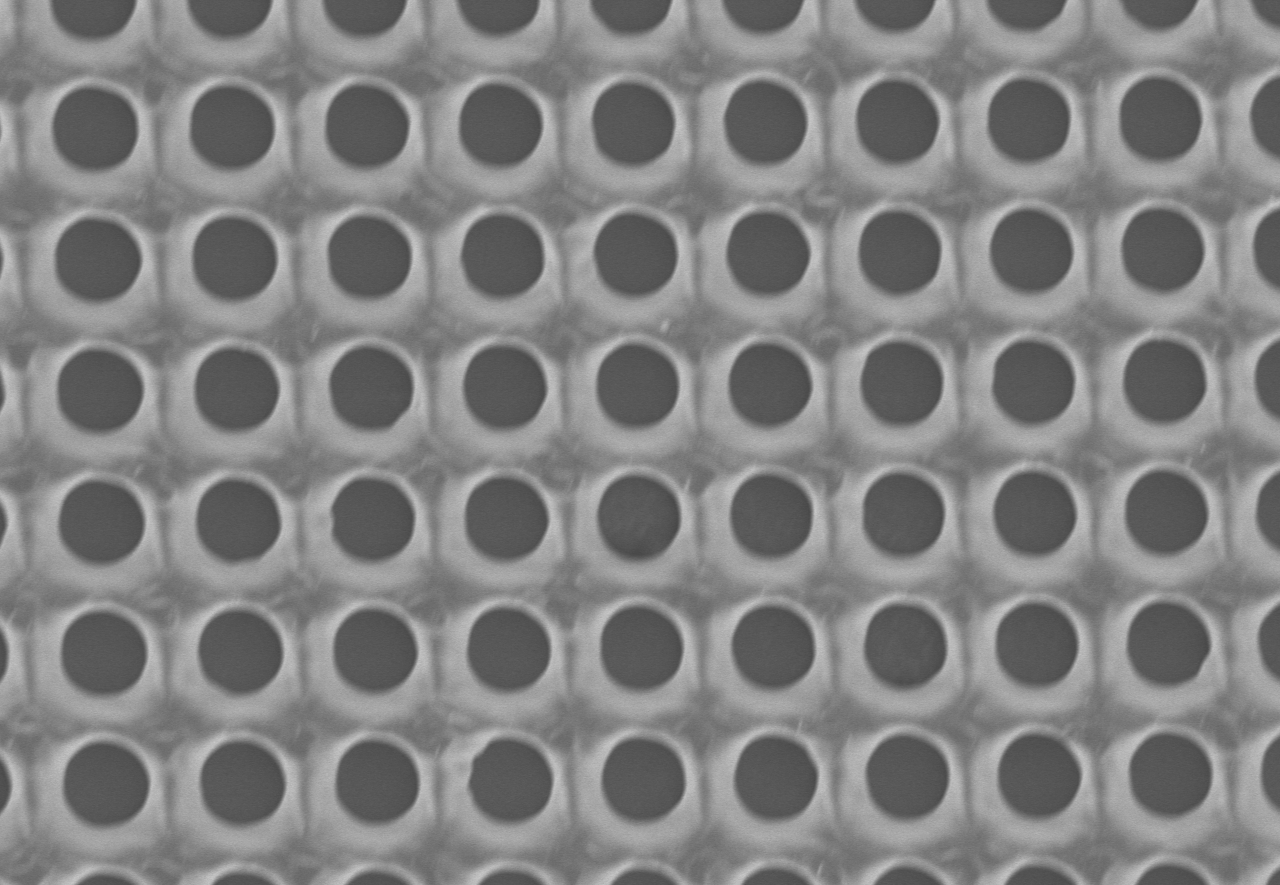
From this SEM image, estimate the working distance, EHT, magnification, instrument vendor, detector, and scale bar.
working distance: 8.2 mm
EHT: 5 kV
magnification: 1.2 K X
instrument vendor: Hitachi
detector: SE(U)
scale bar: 40000 nm